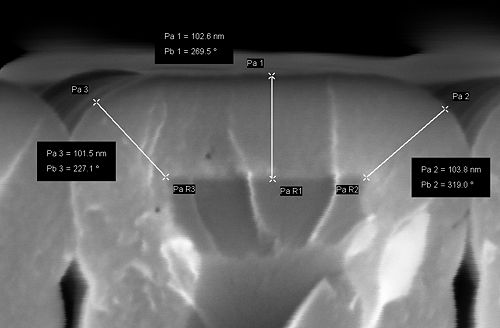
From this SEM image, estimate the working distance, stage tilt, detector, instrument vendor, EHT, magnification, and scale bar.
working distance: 3.4 mm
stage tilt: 0°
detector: InLens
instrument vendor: Zeiss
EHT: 5 kV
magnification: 604.46 K X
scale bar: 20 nm